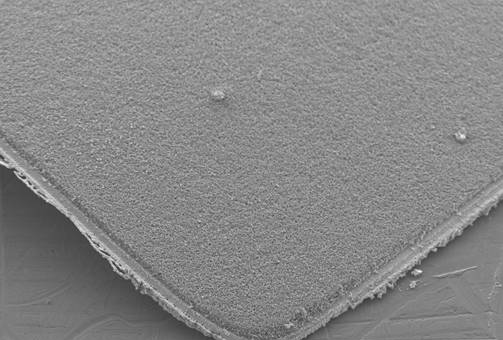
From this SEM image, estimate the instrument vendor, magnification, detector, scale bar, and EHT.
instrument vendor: JEOL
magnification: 0.1 K X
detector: SEI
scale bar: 200000 nm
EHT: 15 kV